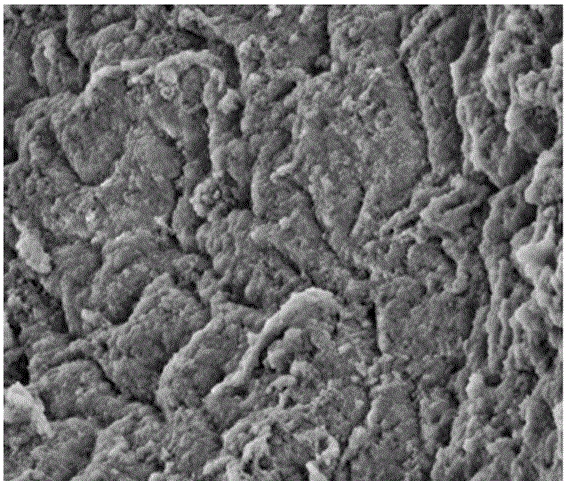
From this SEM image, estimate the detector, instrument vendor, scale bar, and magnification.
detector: ETD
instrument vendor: FEI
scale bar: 5000 nm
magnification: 20 K X